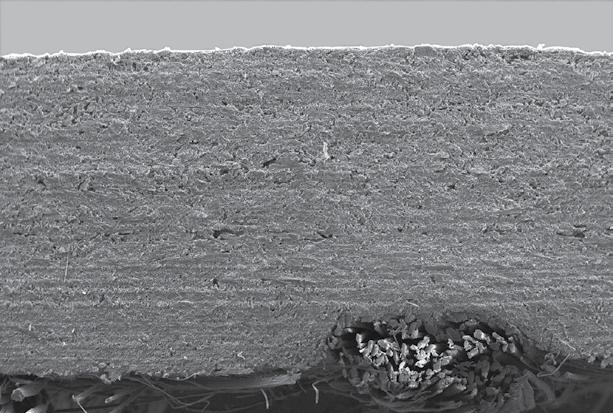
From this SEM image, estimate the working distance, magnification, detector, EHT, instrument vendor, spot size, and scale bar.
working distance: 12.2 mm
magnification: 0.03 K X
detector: SE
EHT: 16 kV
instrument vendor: FEI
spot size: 4.2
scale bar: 1000000 nm